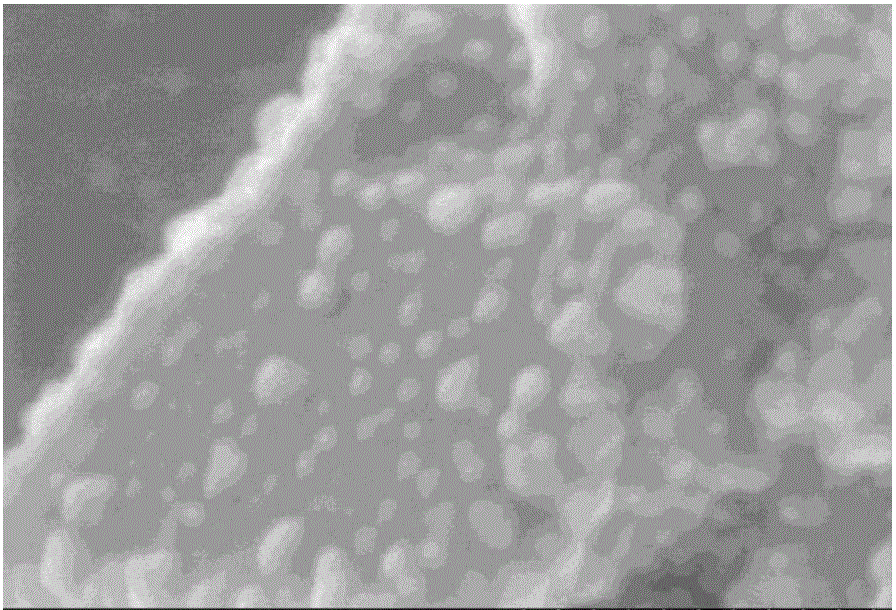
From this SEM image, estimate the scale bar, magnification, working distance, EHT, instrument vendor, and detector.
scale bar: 200 nm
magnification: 250 K X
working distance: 9 mm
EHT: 3 kV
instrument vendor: Hitachi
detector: SE(M)